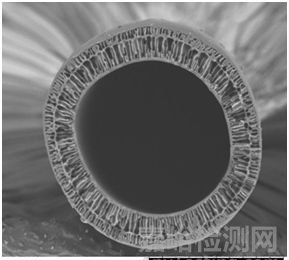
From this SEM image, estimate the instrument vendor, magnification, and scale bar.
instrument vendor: Hitachi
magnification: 0.18 K X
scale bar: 500000 nm